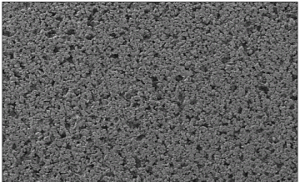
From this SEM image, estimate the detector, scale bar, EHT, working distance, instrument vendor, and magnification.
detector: LEI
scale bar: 100000 nm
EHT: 5 kV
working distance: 8.1 mm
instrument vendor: JEOL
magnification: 0.05 K X